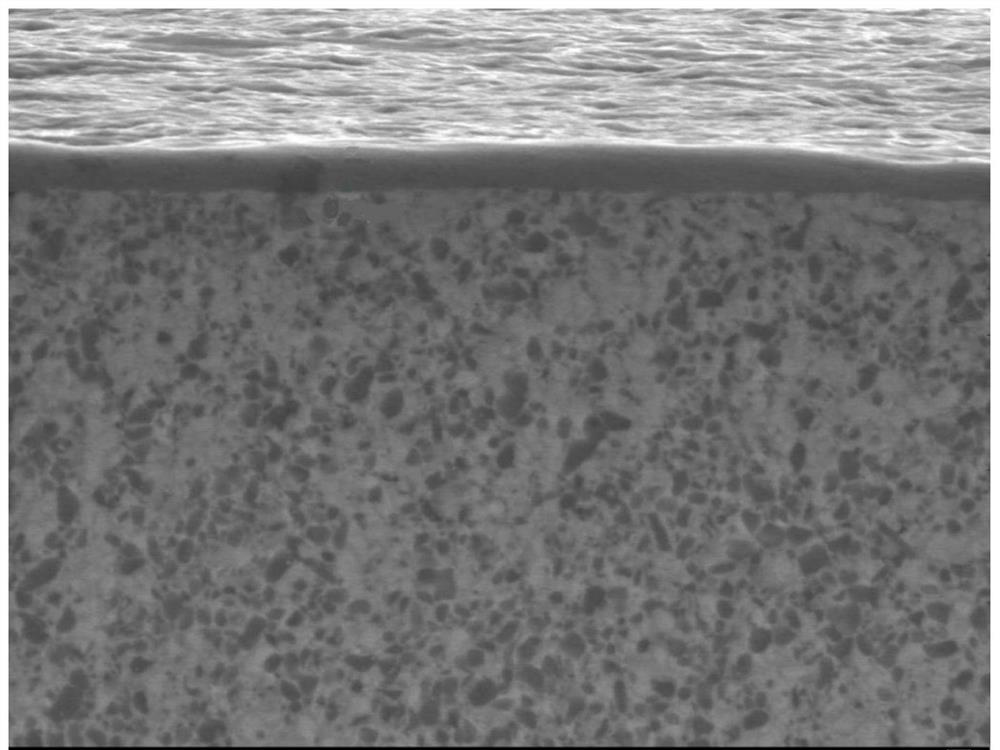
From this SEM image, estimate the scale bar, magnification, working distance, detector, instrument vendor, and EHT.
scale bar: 1000 nm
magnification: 3 K X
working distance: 14.7 mm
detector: LEI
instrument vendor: JEOL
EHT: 20 kV